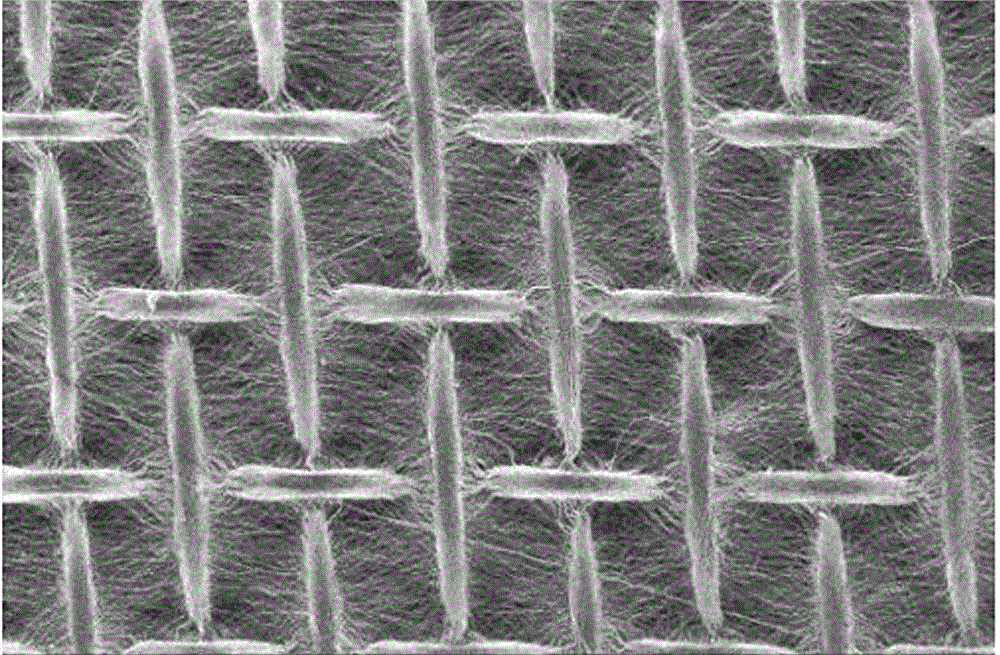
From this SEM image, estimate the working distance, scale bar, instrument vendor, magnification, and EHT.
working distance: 11.5 mm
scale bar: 1000000 nm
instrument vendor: Hitachi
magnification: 0.05 K X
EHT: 20 kV